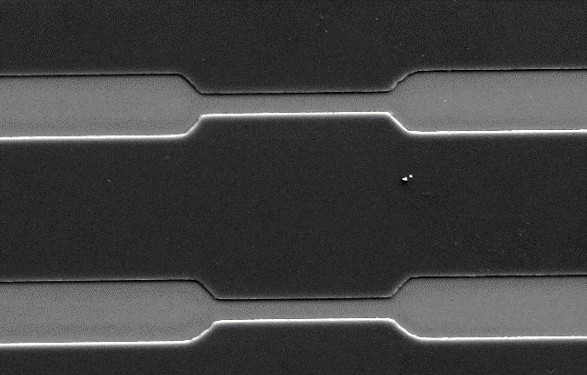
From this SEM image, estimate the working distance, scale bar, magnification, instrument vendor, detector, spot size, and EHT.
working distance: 7.5 mm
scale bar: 20000 nm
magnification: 1.084 K X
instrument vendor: FEI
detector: SE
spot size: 3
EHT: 10 kV